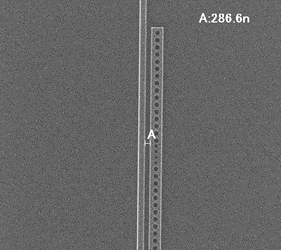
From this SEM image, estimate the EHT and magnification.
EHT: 50 kV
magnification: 8 K X